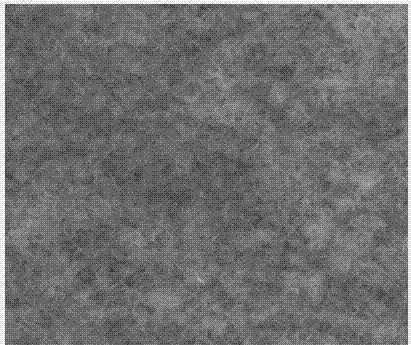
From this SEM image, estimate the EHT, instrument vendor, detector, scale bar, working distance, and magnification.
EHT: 10 kV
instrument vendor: FEI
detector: TLD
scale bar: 1000 nm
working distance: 5.5 mm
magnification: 50 K X